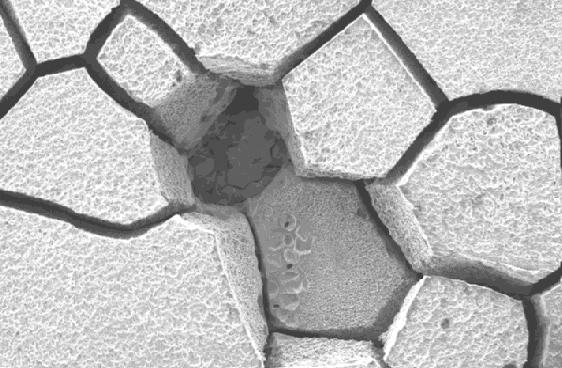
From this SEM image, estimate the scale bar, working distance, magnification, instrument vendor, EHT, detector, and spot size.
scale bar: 200000 nm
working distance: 9.7 mm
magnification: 0.25 K X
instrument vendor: FEI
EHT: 20 kV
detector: SE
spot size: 4